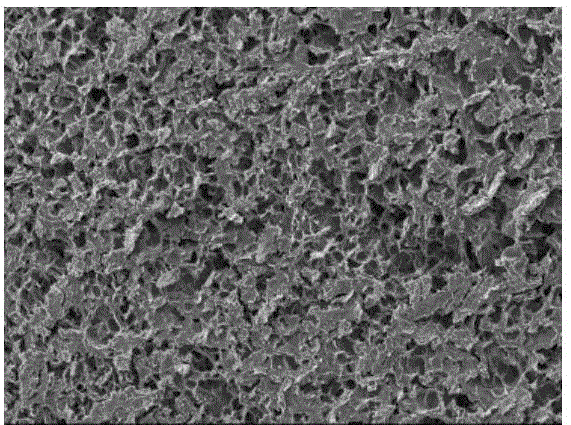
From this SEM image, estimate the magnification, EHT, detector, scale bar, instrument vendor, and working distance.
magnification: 0.2 K X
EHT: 10 kV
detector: SED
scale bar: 100000 nm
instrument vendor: JEOL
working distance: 12.6 mm